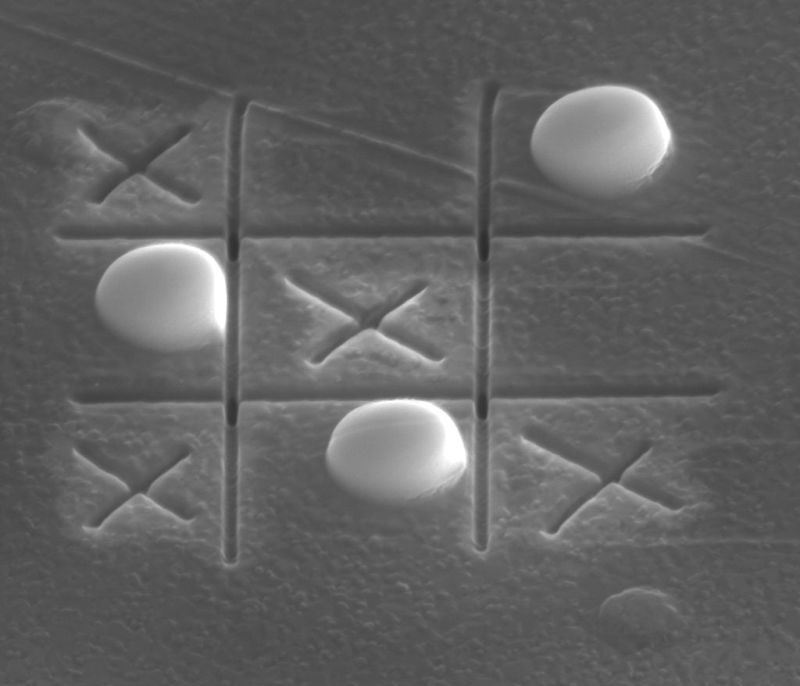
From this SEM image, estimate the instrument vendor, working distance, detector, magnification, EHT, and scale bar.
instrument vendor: FEI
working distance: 4 mm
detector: SE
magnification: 17.5 K X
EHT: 20 kV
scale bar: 2000 nm